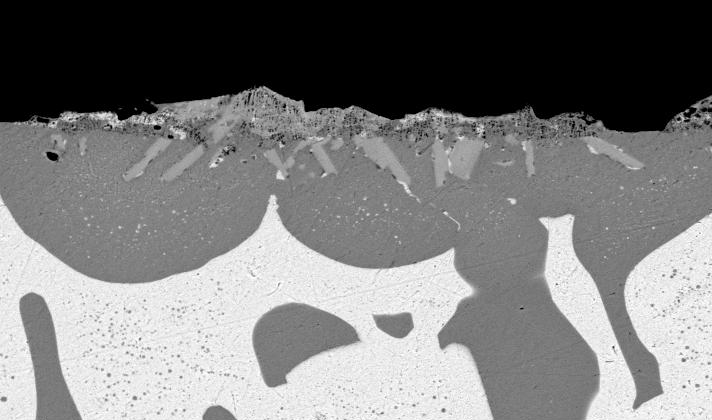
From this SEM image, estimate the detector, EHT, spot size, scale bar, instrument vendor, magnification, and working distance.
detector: BSE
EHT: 20 kV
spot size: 5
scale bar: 20000 nm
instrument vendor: FEI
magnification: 1 K X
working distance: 10.2 mm